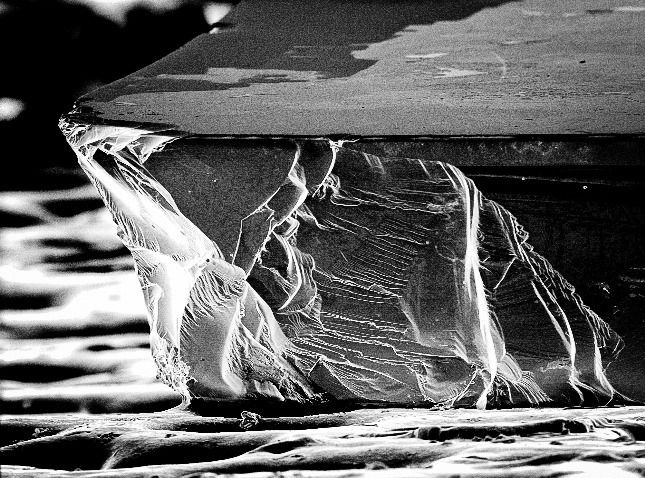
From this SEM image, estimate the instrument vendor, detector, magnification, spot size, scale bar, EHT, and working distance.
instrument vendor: FEI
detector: SE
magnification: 0.181 K X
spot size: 3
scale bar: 100000 nm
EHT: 10 kV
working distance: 18.4 mm